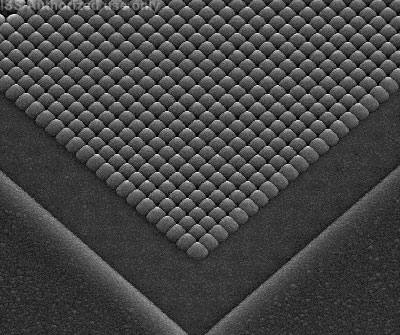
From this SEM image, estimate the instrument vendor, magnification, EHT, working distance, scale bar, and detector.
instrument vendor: FEI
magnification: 8 K X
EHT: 10 kV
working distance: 7.4 mm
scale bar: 10000 nm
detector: ETD